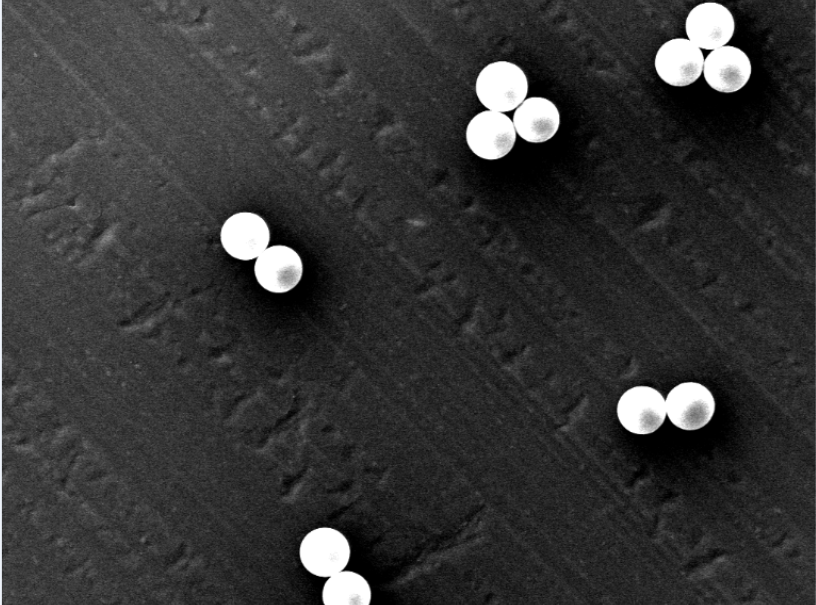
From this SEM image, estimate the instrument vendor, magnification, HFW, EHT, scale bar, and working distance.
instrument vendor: Thermo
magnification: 1.8 K X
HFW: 149 µm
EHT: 15 kV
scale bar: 30000 nm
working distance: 6.392 mm